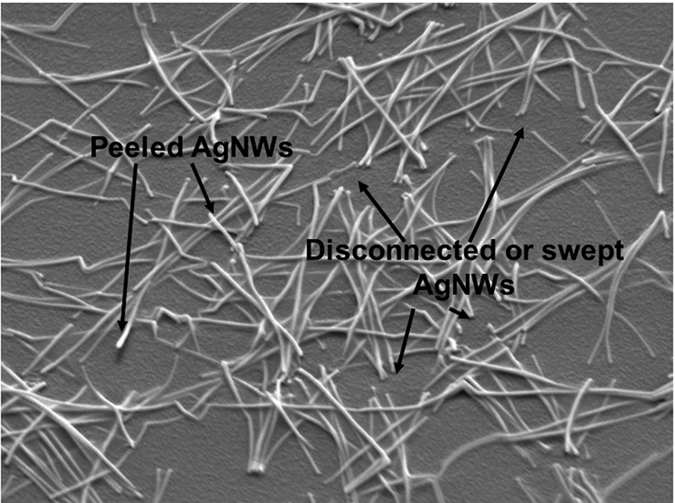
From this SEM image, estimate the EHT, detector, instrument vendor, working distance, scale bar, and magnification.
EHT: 5 kV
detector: SEI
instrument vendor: JEOL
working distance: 15.1 mm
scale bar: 1000 nm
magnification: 20 K X